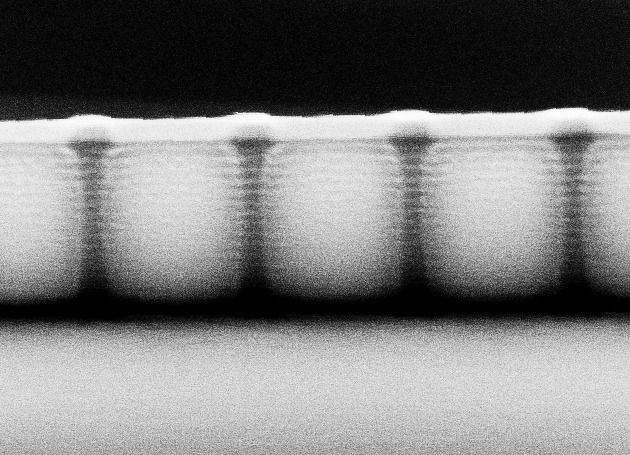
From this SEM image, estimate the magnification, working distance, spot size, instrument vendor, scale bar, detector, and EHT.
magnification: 60.686 K X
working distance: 13.1 mm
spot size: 4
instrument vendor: FEI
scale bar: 500 nm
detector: SE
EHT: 30 kV